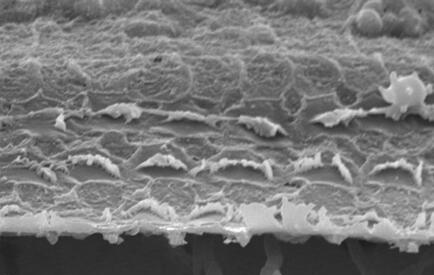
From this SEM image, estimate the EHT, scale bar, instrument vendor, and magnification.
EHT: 20 kV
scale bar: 5500 nm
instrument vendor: JEOL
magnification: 1.5 K X